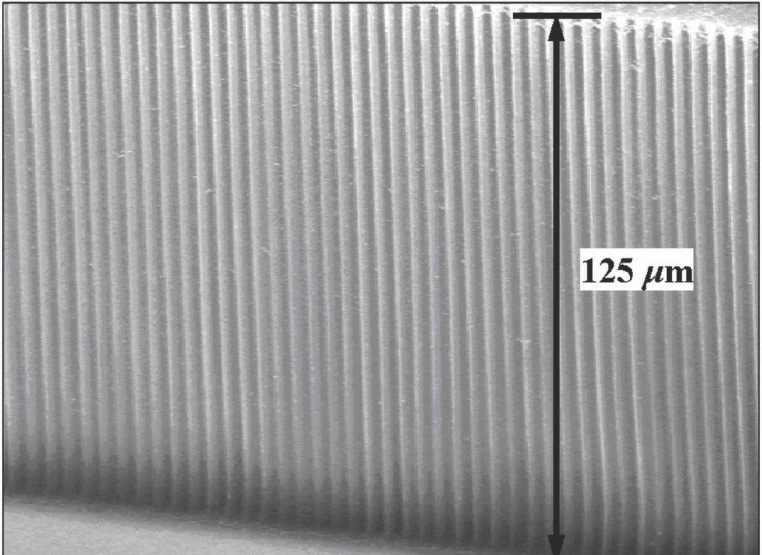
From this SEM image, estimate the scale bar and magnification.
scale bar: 20000 nm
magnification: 1 K X